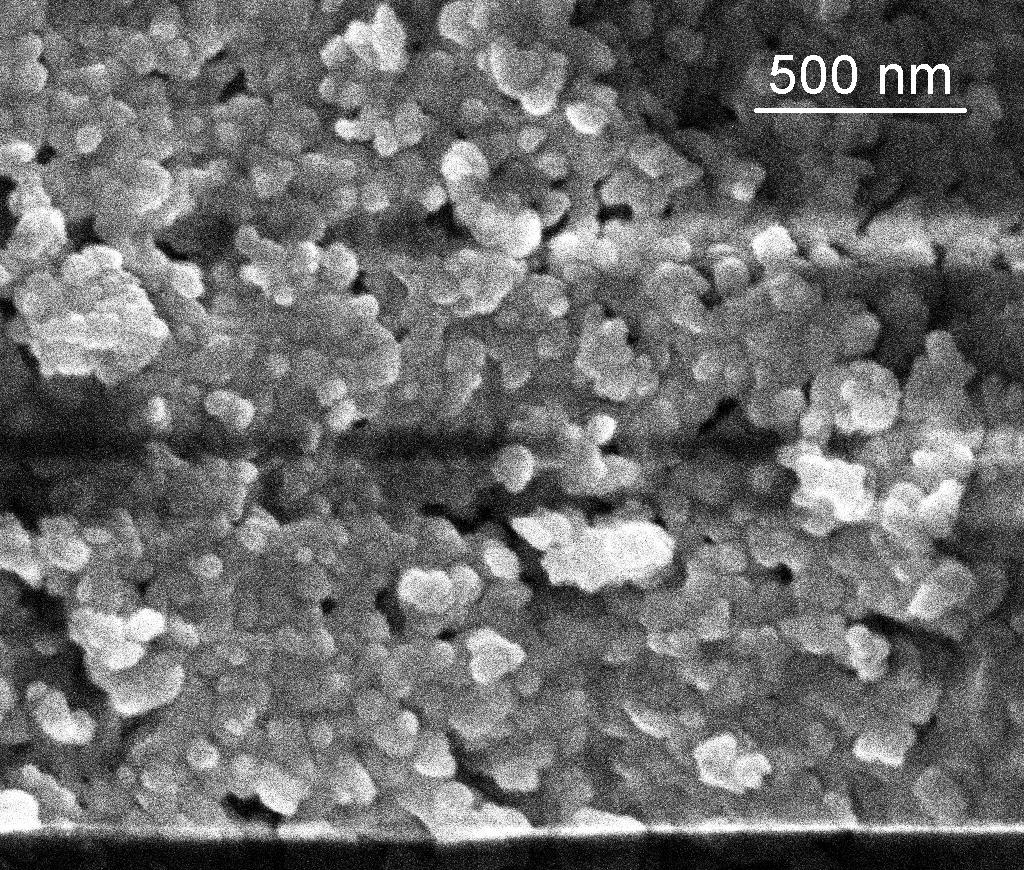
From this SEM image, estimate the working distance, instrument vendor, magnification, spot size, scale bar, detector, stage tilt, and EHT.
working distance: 5.1 mm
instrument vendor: FEI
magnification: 120 K X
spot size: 3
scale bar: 500 nm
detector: TLD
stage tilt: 0°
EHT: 5 kV